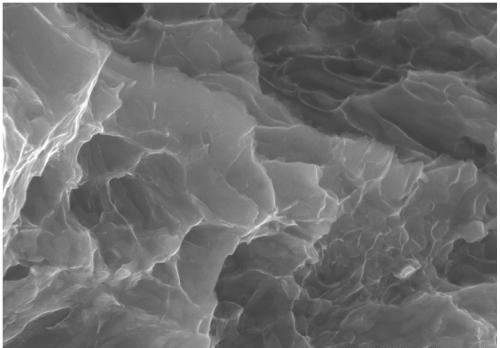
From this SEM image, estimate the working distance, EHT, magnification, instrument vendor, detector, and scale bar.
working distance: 12.8 mm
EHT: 30 kV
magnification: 20 K X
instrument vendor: Hitachi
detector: SE(L)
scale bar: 2000 nm